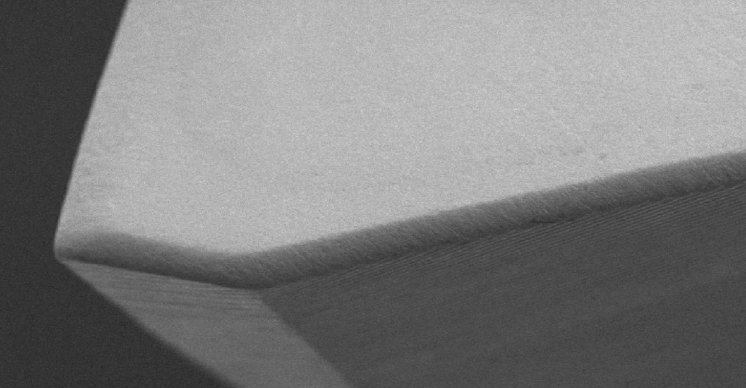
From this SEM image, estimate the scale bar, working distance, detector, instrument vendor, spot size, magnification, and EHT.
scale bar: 100000 nm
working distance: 11.6 mm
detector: SE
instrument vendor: FEI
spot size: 5.5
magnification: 0.2 K X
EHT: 25 kV